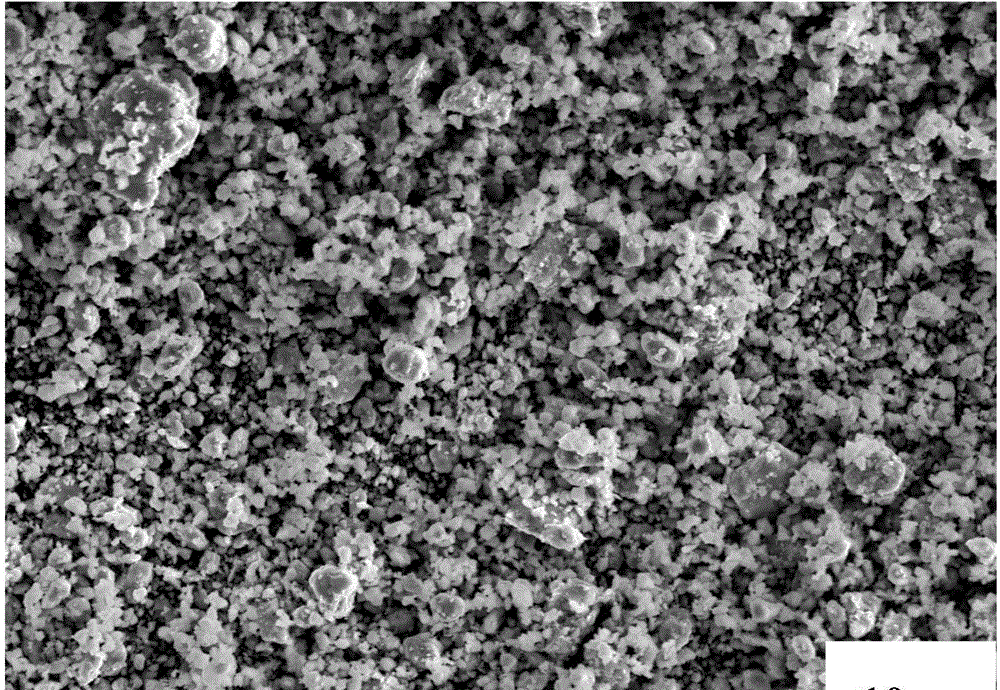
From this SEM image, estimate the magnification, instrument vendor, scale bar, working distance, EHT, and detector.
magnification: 1 K X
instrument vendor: Hitachi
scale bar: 10000 nm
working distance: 11.5 mm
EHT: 20 kV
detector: SE